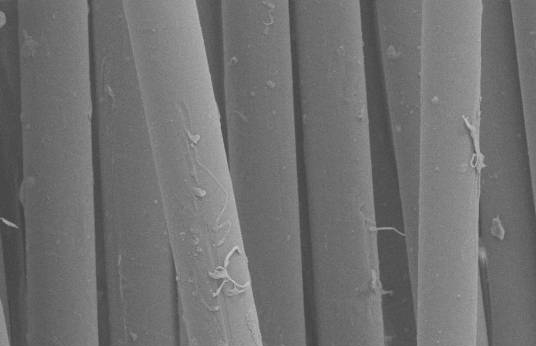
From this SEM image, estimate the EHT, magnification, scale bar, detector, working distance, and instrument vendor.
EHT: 8 kV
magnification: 1 K X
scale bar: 10000 nm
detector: SE1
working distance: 20 mm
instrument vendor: Zeiss